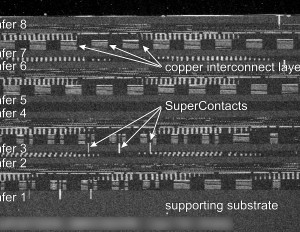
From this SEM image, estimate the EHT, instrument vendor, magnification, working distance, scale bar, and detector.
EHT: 5 kV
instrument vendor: Hitachi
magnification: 0.5 K X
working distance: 8.7 mm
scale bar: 100000 nm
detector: SE(M)